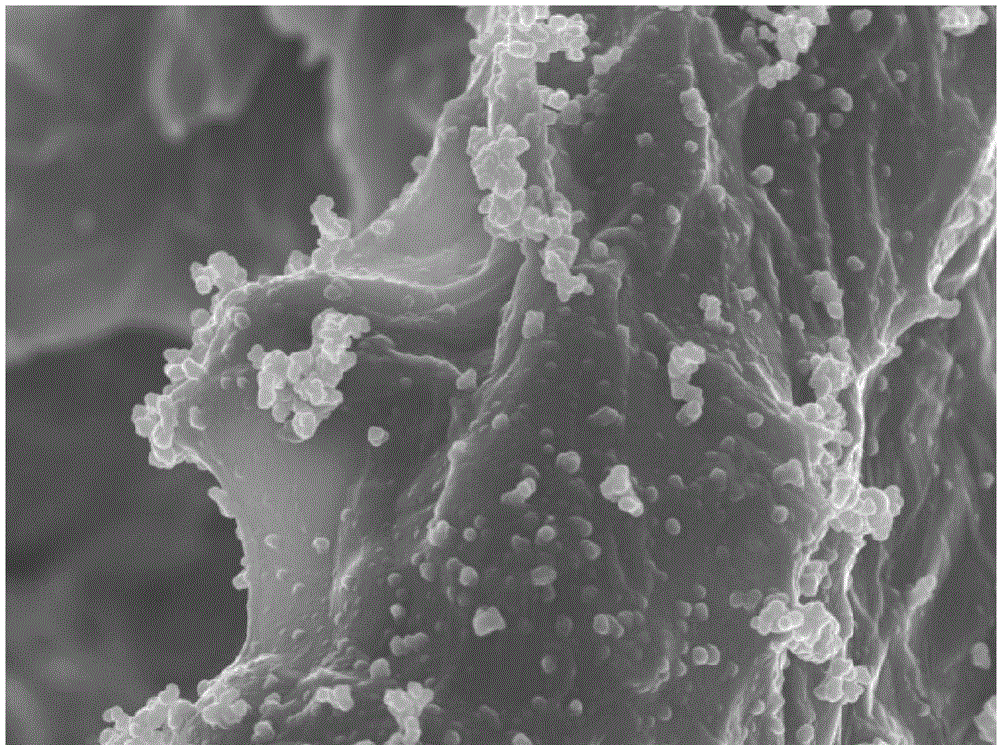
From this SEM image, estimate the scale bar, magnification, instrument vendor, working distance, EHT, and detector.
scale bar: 1000 nm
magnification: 5 K X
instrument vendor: JEOL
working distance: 10 mm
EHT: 10 kV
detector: SEI